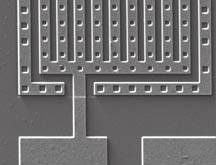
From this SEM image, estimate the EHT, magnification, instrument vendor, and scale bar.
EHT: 10 kV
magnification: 1 K X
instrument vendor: JEOL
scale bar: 20000 nm